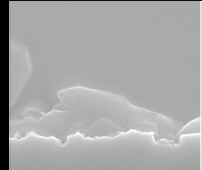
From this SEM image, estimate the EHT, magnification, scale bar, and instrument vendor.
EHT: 15 kV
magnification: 30 K X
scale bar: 1000 nm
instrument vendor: JEOL